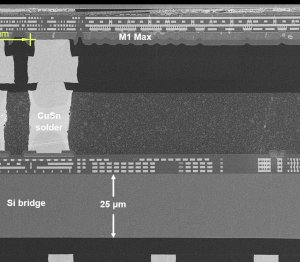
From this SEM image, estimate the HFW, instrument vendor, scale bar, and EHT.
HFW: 151.1 µm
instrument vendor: Zeiss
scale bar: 20000 nm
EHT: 5 kV